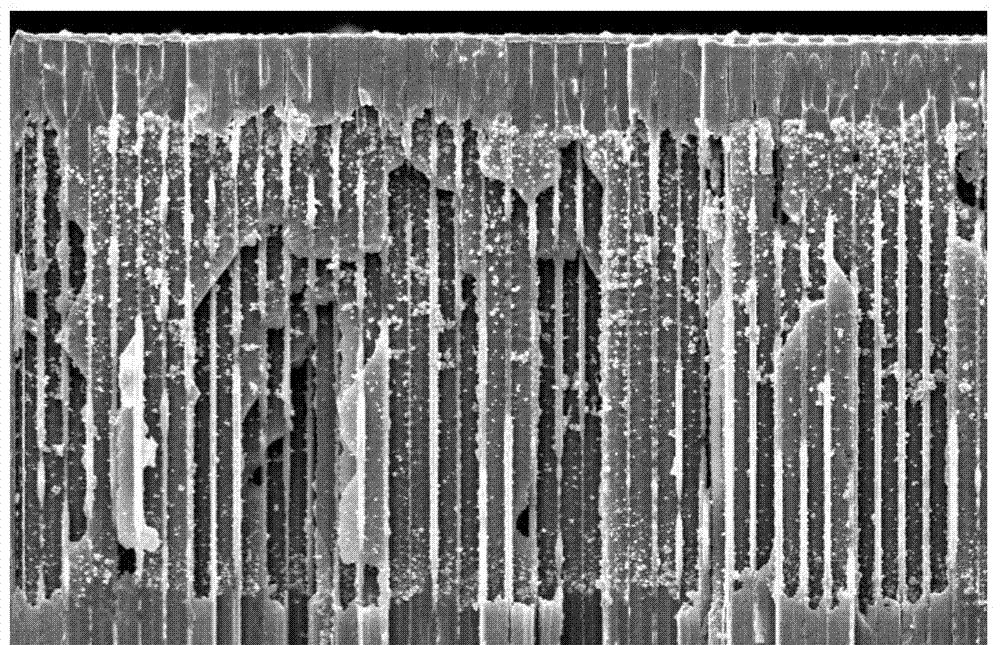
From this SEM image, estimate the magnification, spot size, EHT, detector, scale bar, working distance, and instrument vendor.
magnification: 0.5 K X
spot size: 3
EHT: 20 kV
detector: SE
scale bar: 50000 nm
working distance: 7.8 mm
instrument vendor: FEI